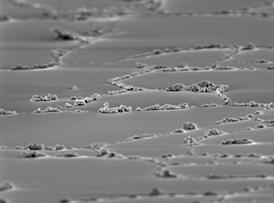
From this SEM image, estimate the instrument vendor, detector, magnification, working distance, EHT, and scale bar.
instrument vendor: JEOL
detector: SEI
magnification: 0.5 K X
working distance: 12 mm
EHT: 15 kV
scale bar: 10000 nm